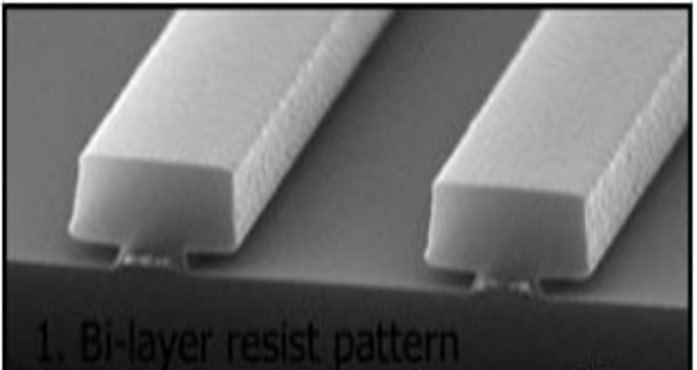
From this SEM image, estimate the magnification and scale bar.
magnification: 75 K X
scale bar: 1000 nm